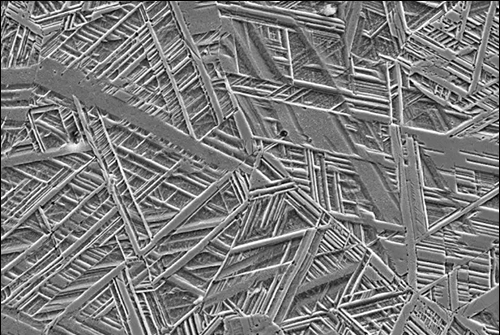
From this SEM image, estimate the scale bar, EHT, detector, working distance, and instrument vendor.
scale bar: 10000 nm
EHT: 15 kV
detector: SE2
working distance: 14.7 mm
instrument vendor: Zeiss